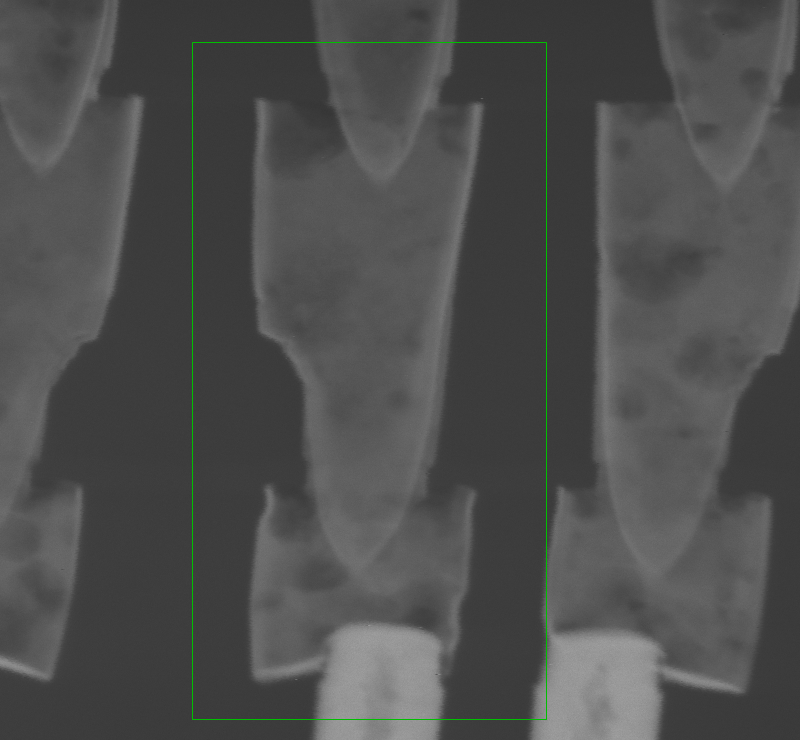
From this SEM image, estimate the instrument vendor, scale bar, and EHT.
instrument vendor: FEI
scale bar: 300 nm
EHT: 200 kV